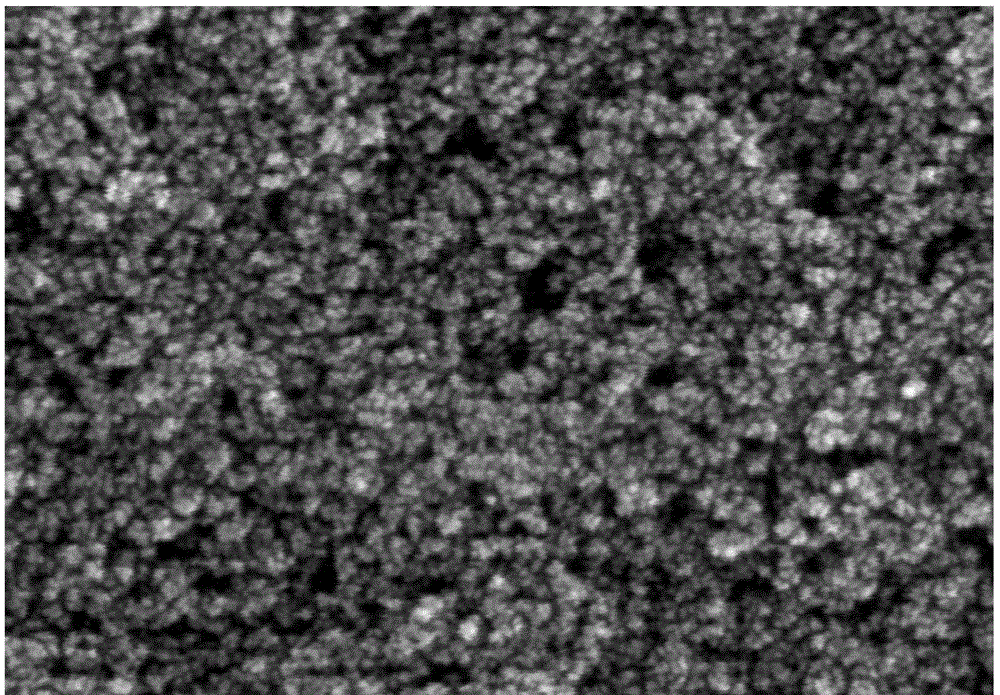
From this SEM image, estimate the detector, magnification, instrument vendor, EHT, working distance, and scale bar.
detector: SE(U)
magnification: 200 K X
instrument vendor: Hitachi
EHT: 5 kV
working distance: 6.7 mm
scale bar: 200 nm